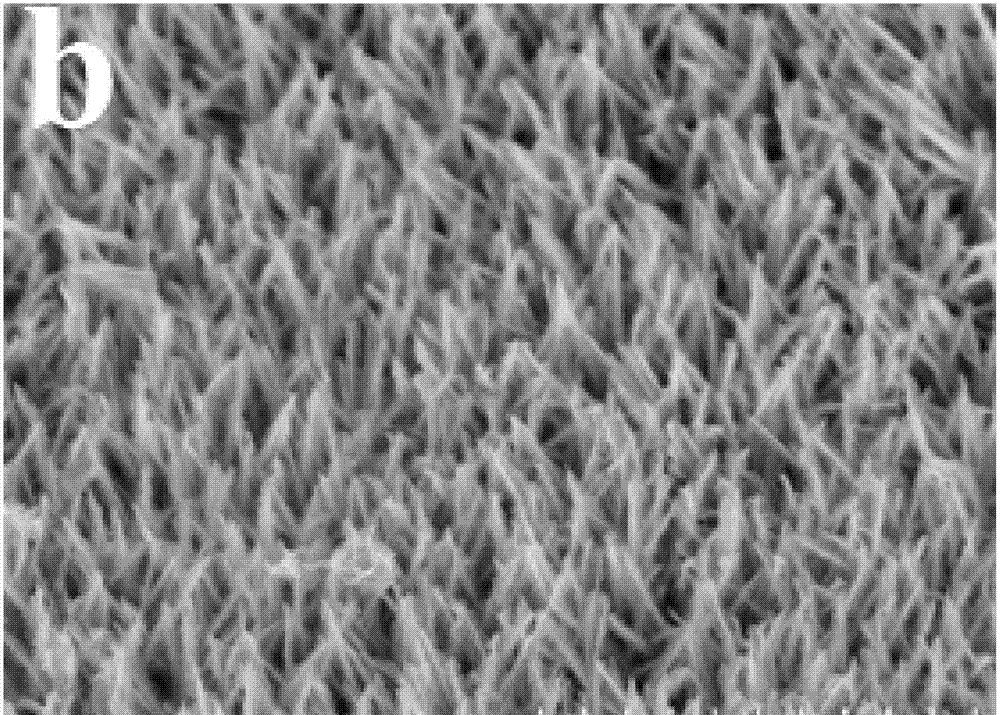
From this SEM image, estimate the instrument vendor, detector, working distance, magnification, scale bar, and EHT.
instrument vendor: Hitachi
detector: SE(M)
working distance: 18.3 mm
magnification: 11 K X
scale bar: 5000 nm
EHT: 5 kV